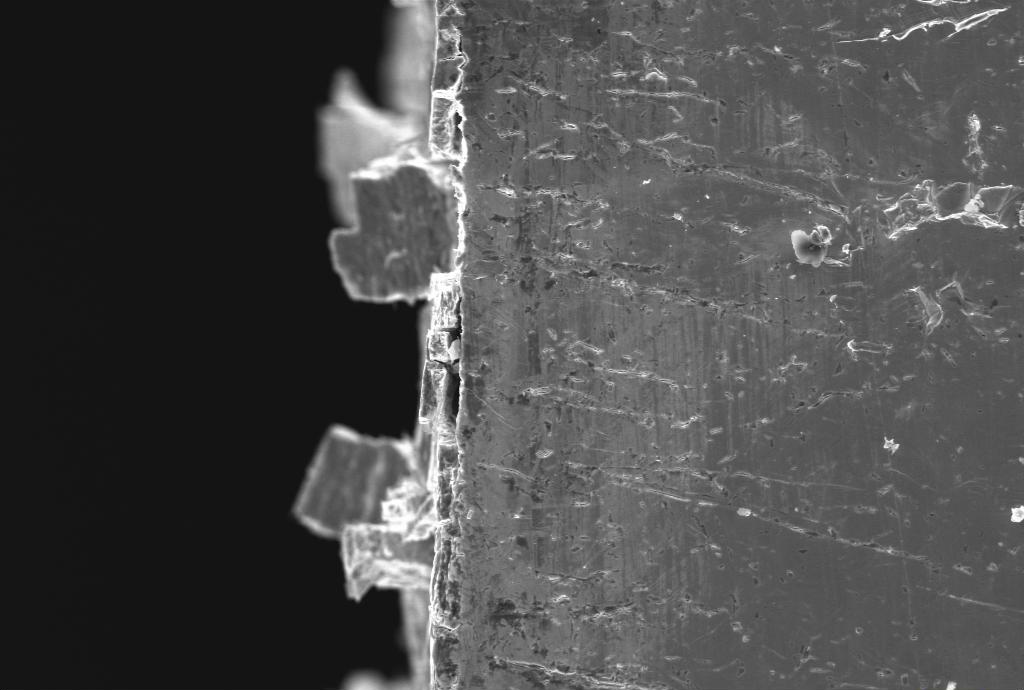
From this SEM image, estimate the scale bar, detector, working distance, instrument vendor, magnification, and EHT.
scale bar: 20000 nm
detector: SE1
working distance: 30 mm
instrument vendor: Zeiss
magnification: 0.678 K X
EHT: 20 kV